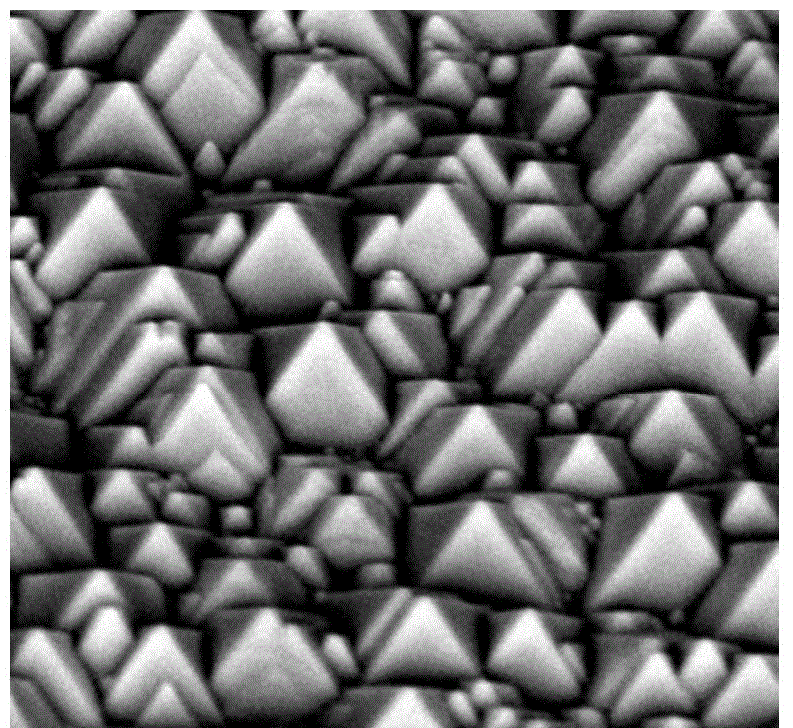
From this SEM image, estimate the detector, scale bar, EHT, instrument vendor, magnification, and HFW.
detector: BSD Full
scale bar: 5000 nm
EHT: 10 kV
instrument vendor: Phenom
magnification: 12.5 K X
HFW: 21.5 µm